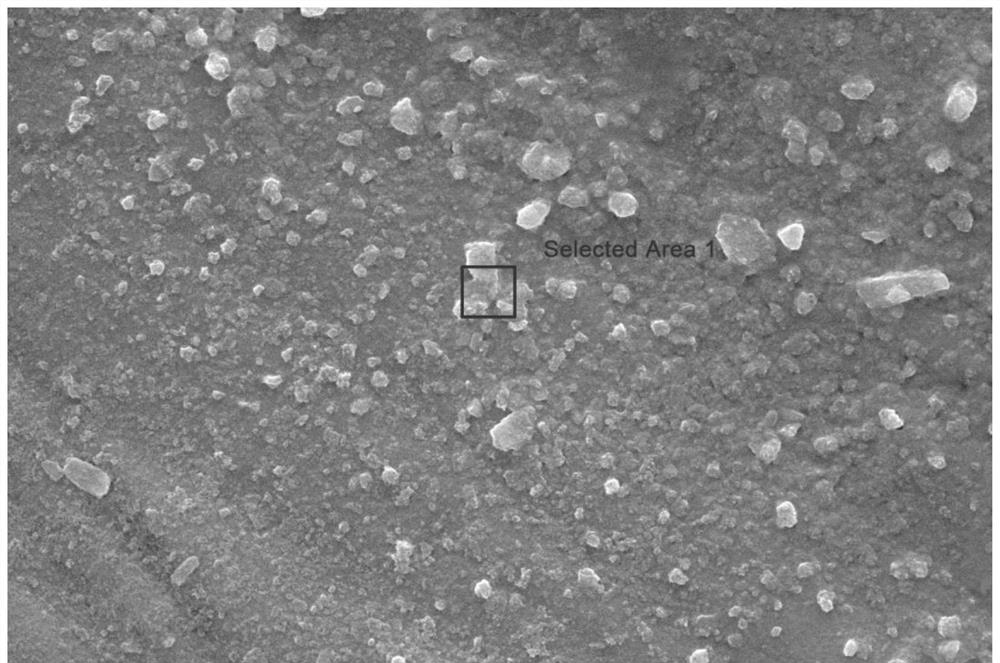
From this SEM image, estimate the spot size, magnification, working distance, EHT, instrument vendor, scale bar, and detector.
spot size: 3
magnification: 5 K X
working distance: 11.3 mm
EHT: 20 kV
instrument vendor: FEI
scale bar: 10000 nm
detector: SE